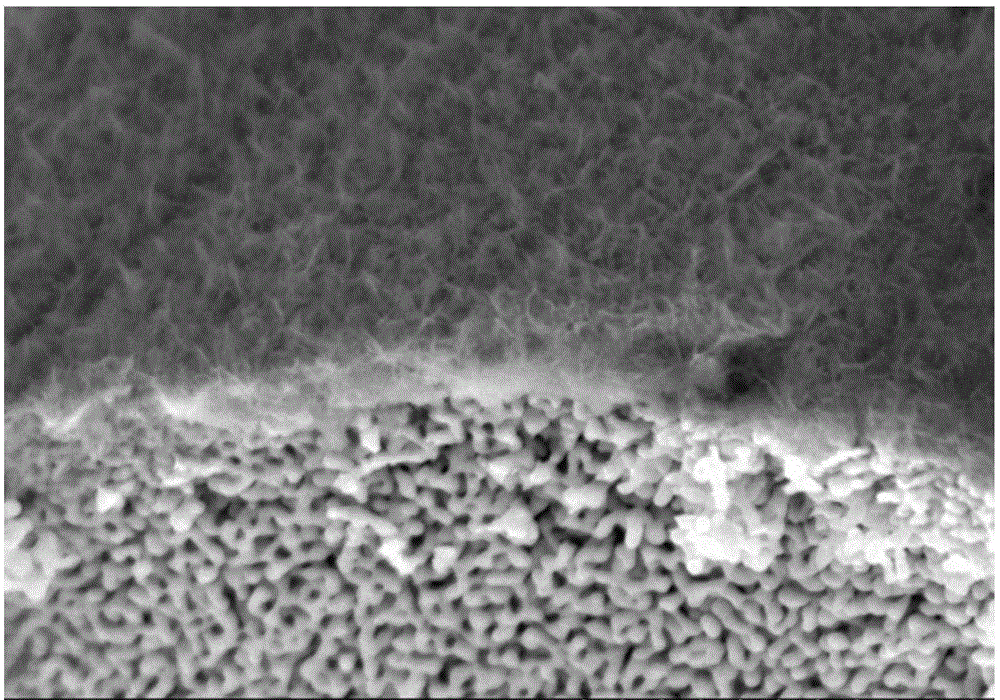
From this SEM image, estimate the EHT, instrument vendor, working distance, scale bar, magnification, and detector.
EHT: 5 kV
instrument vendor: Hitachi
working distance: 8.3 mm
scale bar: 1000 nm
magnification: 50 K X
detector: SE(M,LA0)